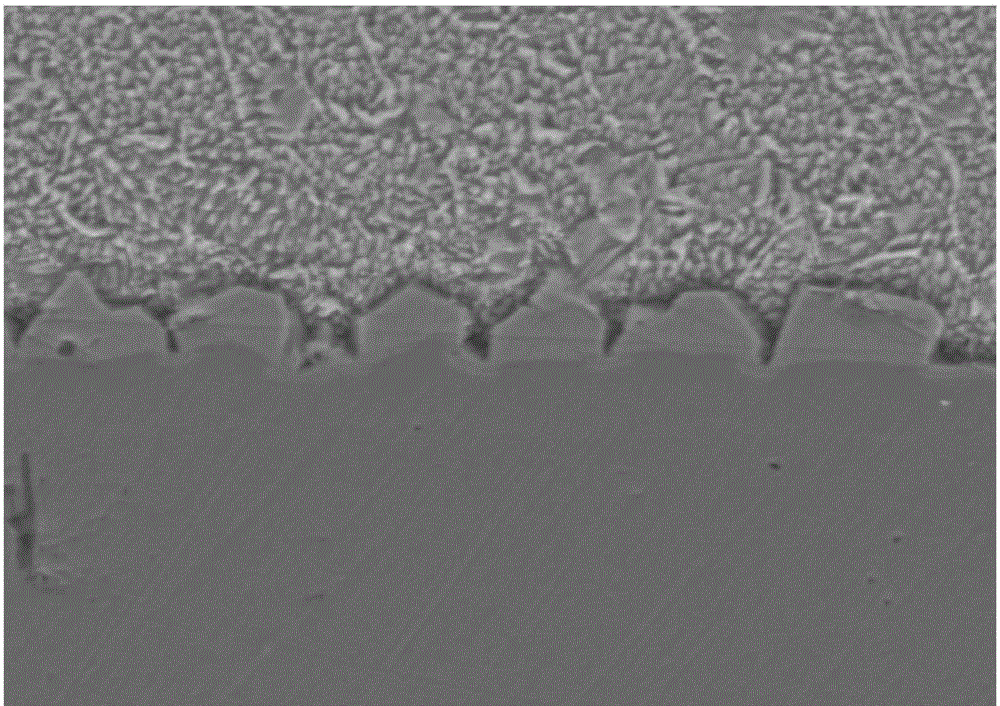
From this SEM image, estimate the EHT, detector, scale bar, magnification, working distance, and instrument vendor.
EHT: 15 kV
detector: SE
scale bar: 20000 nm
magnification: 2 K X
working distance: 14.7 mm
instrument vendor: Hitachi